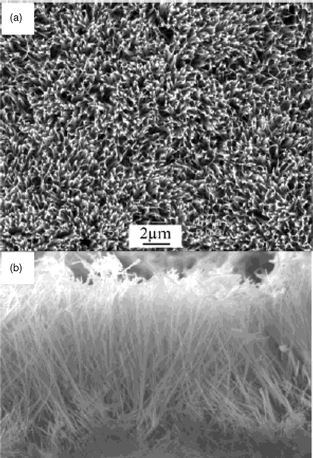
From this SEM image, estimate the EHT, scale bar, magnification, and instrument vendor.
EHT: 15 kV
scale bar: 10000 nm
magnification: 3 K X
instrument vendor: JEOL